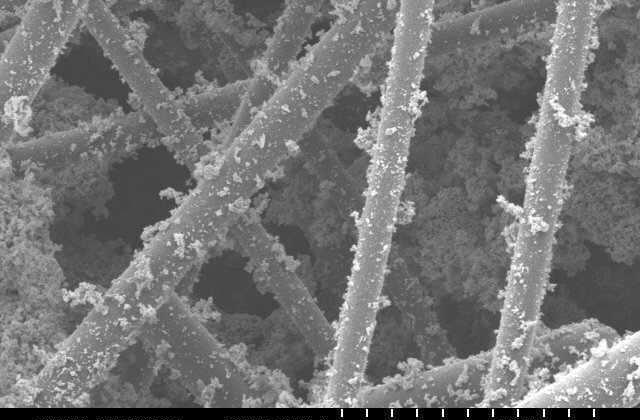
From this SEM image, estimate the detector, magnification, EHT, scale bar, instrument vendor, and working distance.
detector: SE(M)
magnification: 1 K X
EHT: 5 kV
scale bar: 50000 nm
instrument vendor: Hitachi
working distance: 11.4 mm